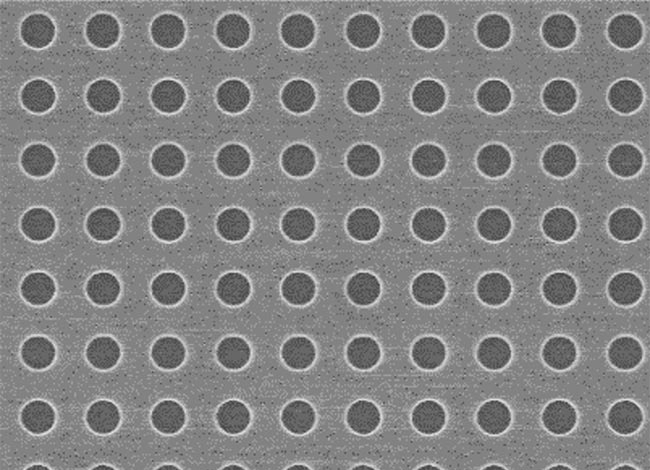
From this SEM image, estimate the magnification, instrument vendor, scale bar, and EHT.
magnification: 20 K X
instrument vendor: JEOL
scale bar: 1500 nm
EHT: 10 kV